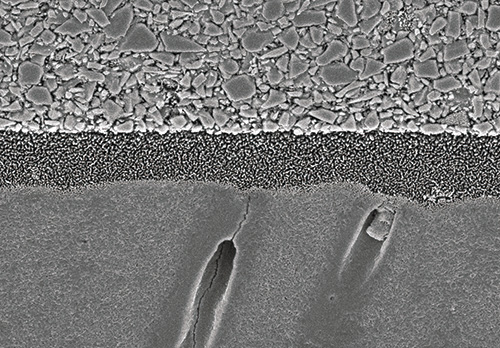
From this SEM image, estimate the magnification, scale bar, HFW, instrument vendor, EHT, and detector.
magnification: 5 K X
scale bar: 5000 nm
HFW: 24 µm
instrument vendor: JEOL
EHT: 5 kV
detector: A+B